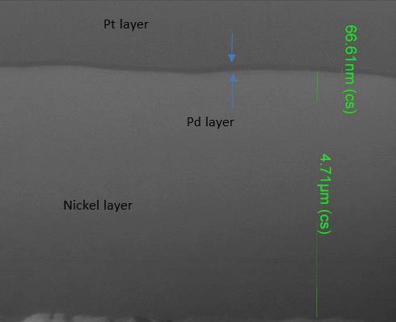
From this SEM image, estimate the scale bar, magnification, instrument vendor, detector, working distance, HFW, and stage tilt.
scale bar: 3000 nm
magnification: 50 K X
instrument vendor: FEI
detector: ETD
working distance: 30.1 mm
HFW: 5.97 µm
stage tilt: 0°